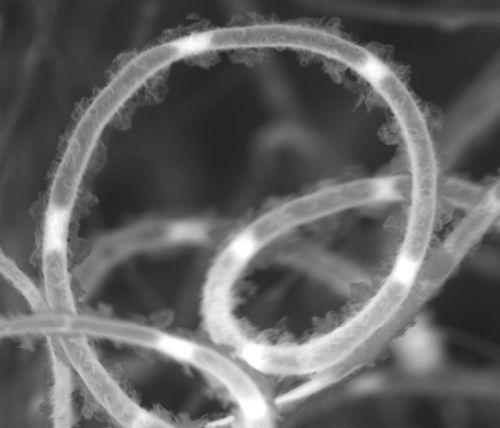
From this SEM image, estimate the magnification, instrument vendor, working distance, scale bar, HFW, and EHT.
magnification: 5.4 K X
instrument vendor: FEI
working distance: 9.6 mm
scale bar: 10000 nm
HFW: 50.07 µm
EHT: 20 kV